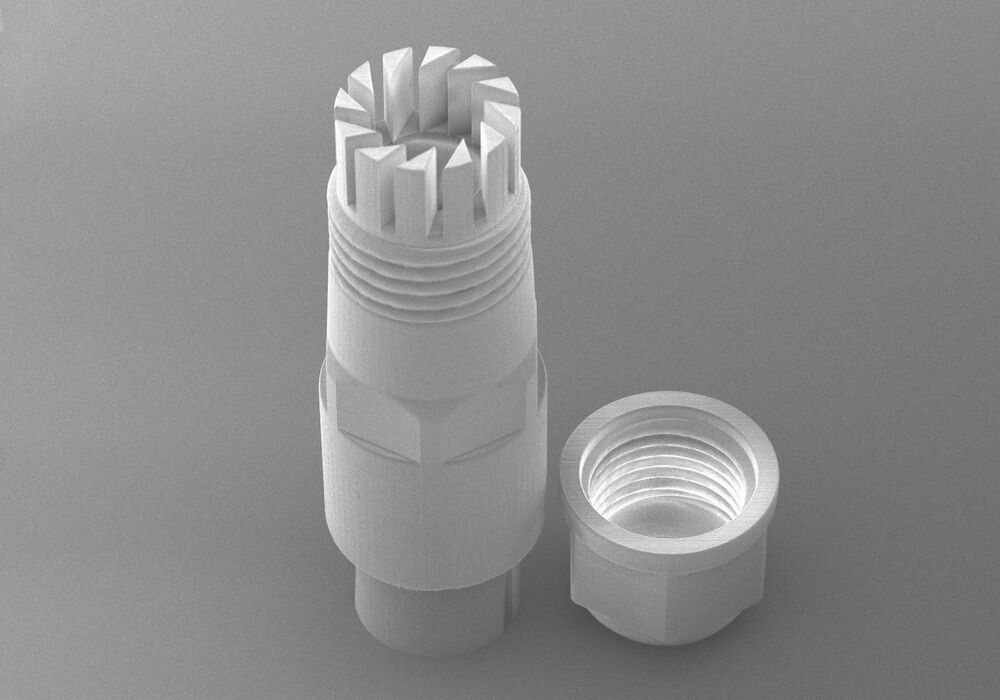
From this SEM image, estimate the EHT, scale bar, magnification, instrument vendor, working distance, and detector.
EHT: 7 kV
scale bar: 1000000 nm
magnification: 0.045 K X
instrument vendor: Hitachi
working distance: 9.1 mm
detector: SE